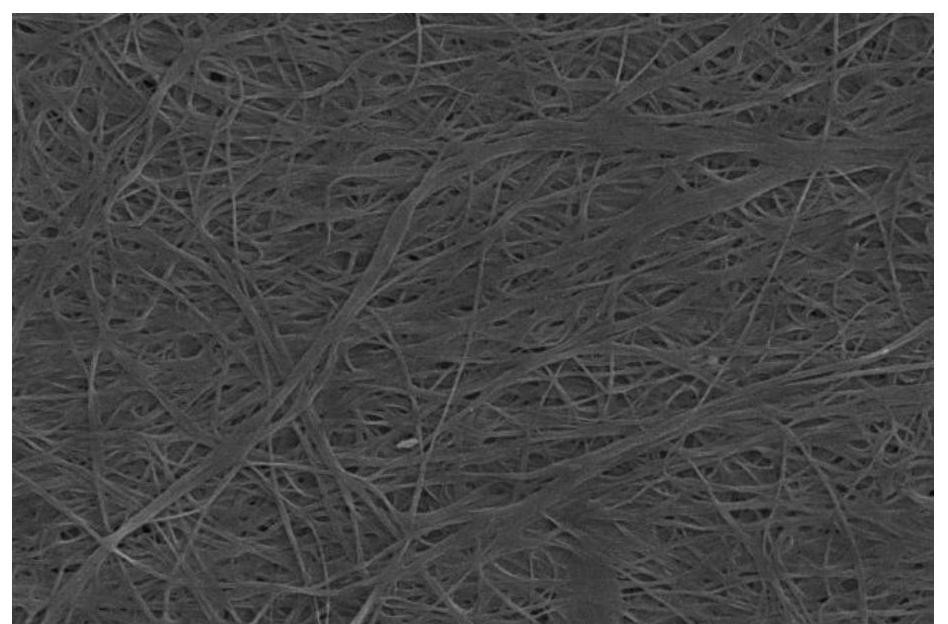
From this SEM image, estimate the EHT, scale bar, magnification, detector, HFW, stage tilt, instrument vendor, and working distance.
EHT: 5 kV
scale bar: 2000 nm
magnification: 20 K X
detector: ETD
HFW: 10.4 µm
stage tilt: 0°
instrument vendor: Thermo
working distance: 4.5 mm